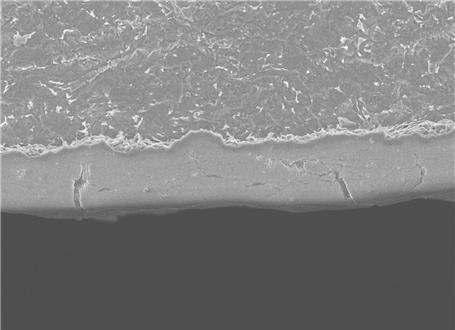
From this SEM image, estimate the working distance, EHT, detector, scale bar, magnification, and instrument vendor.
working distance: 6 mm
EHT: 5 kV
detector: SEI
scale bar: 1000 nm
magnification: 5 K X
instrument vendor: JEOL